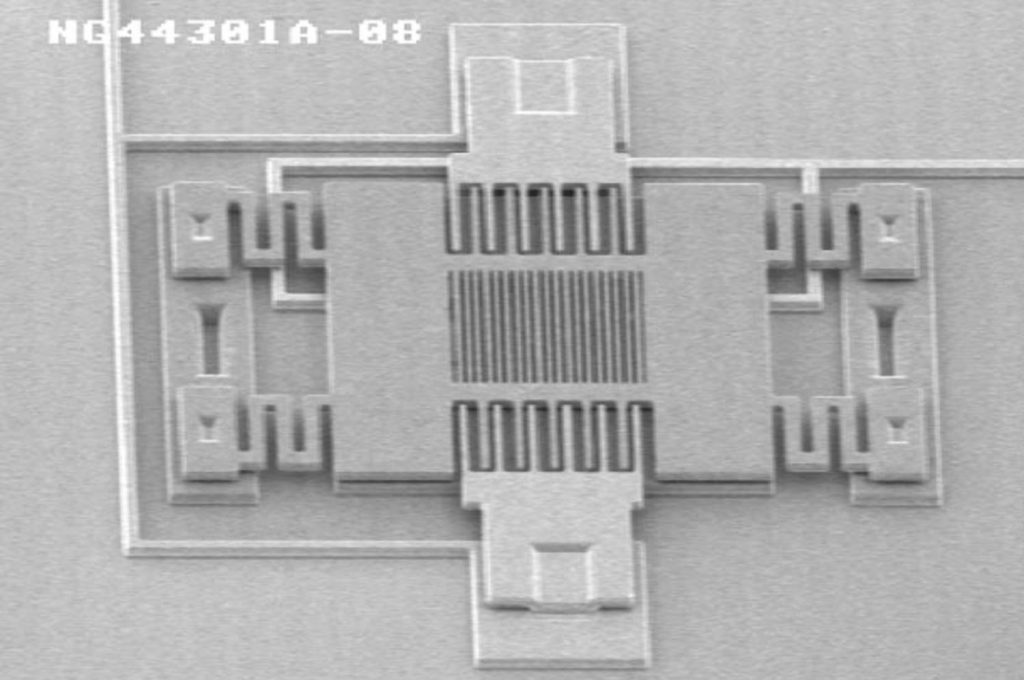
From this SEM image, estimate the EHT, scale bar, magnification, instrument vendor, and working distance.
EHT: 2 kV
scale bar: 10000 nm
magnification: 1.6 K X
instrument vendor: JEOL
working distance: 10 mm